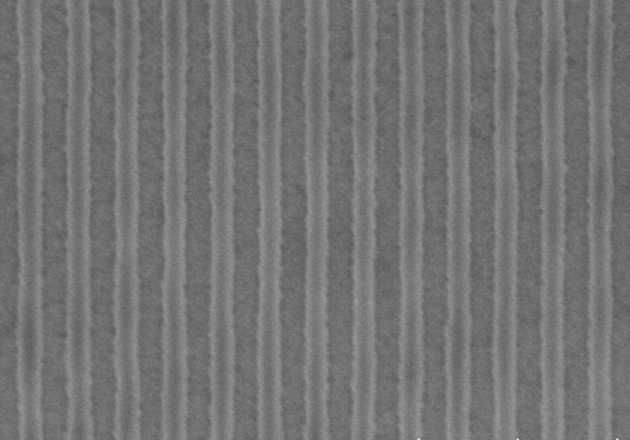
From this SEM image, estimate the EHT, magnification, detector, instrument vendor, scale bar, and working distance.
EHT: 2 kV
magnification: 40 K X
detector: SE(UL)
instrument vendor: Hitachi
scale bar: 1000 nm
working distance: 9.2 mm